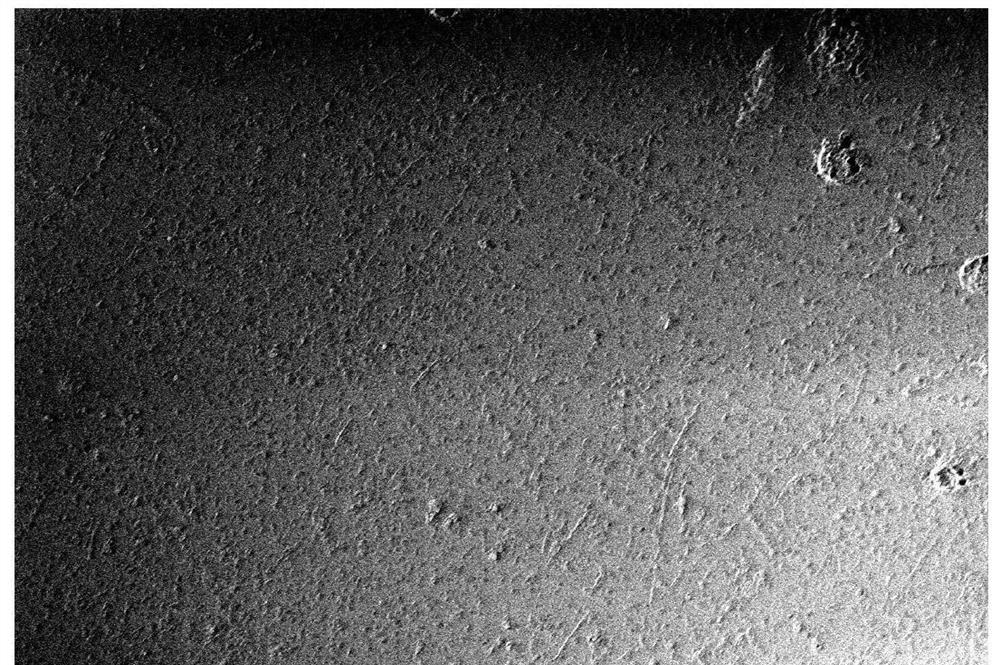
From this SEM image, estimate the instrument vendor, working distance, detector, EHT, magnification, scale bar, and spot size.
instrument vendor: JEOL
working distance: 18 mm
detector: SEI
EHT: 2 kV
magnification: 0.2 K X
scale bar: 100000 nm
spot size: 56